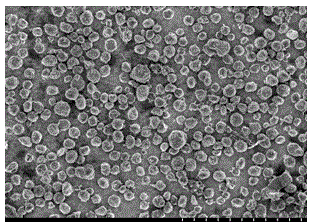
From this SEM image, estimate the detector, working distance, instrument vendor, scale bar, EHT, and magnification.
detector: SE(M)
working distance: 10.2 mm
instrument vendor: Hitachi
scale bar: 100000 nm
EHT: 3 kV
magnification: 0.5 K X